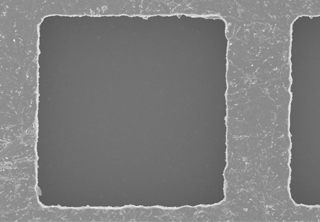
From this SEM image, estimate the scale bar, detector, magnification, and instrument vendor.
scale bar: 10000 nm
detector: SE(UL)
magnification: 4 K X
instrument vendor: Hitachi